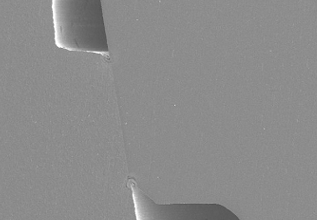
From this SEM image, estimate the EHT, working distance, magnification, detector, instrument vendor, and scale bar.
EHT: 10 kV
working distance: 15.1 mm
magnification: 0.1 K X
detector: SE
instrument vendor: JEOL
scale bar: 500000 nm